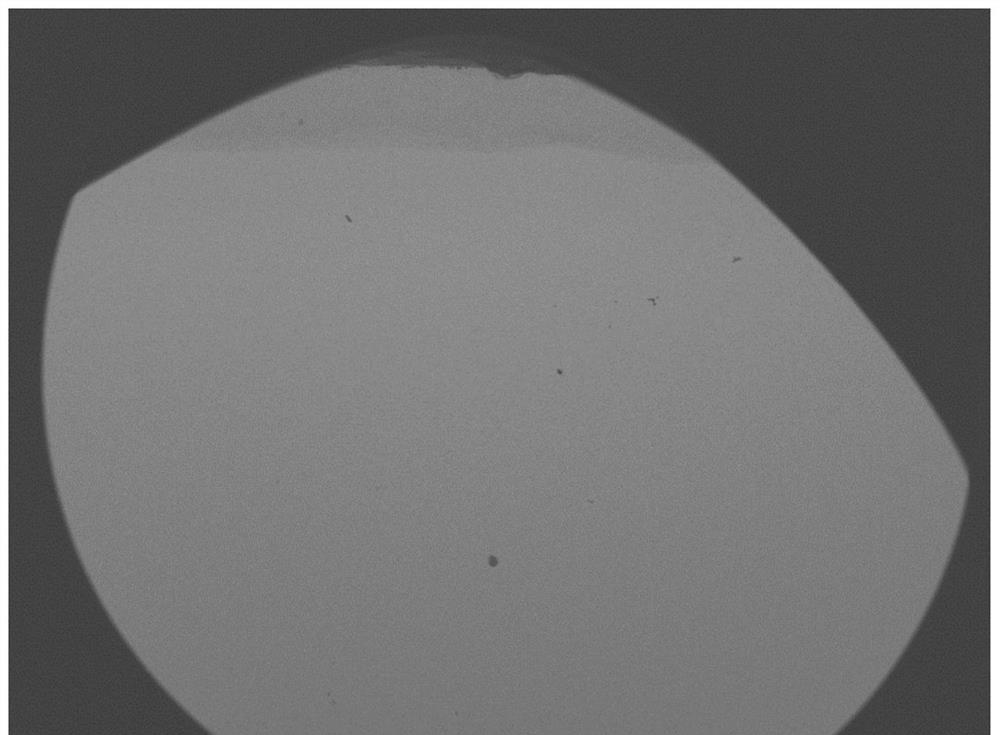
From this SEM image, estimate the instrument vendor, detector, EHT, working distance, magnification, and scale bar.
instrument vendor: JEOL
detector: COMPO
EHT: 15 kV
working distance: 10.9 mm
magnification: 0.02 K X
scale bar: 1000000 nm